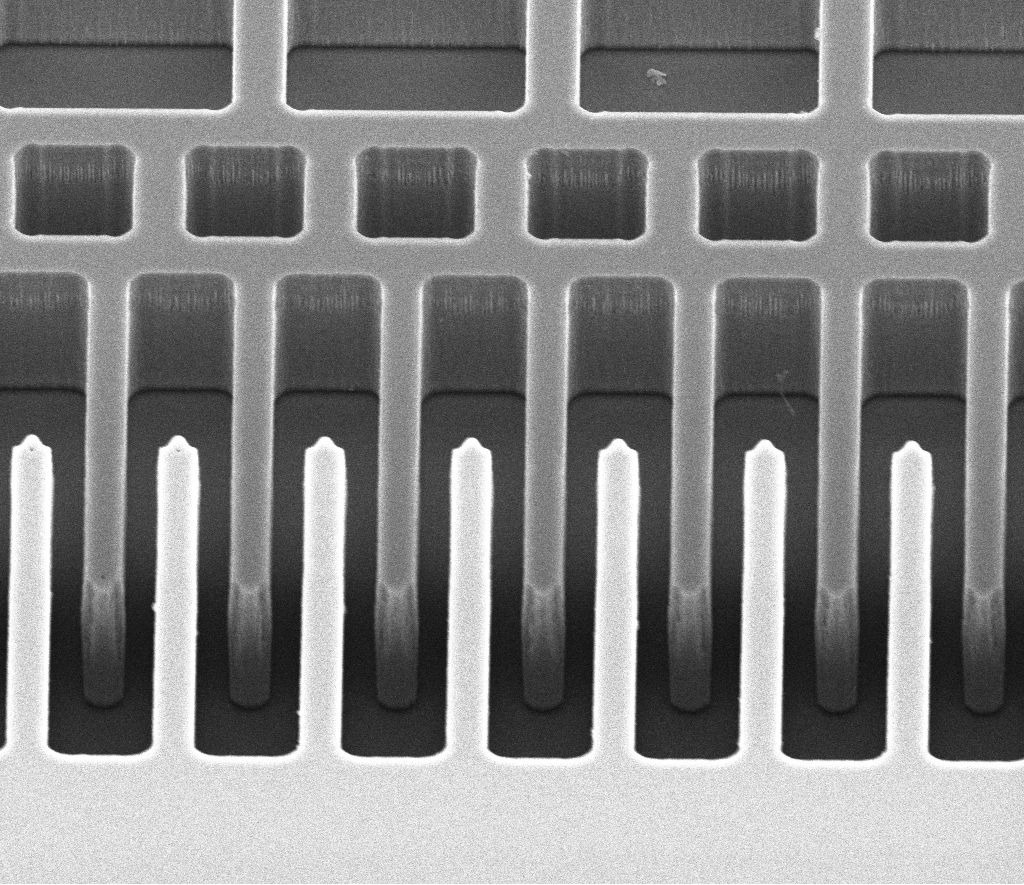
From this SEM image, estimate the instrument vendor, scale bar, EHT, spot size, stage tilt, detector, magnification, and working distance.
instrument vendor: FEI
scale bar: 20000 nm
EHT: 10 kV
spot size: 2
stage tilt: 41°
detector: ETD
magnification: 3 K X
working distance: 20.5 mm